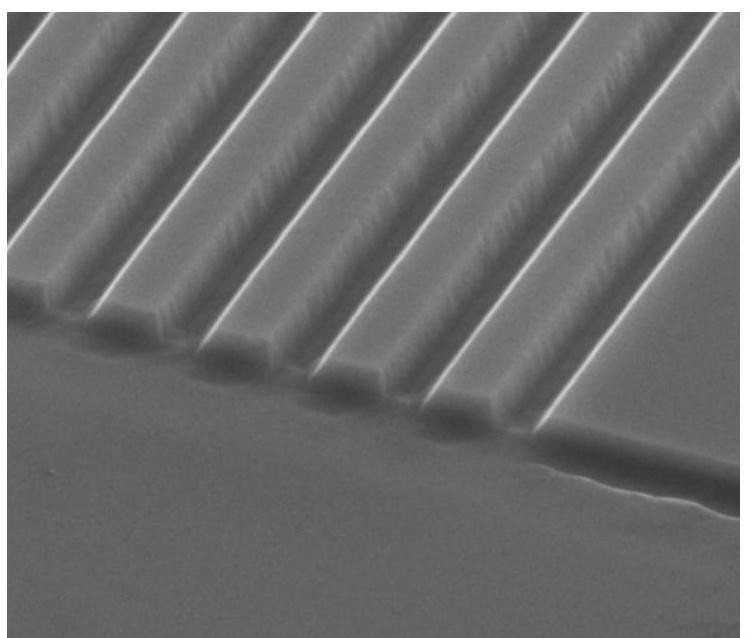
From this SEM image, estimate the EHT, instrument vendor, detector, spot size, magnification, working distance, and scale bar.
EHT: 10 kV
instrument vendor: FEI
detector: ETD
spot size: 4.5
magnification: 50 K X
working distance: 10.6 mm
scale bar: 2000 nm